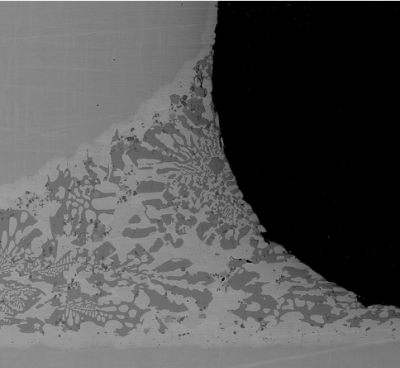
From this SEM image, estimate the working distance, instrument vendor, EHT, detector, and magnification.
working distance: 10.2 mm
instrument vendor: Hitachi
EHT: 20 kV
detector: BSECOMP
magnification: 0.25 K X